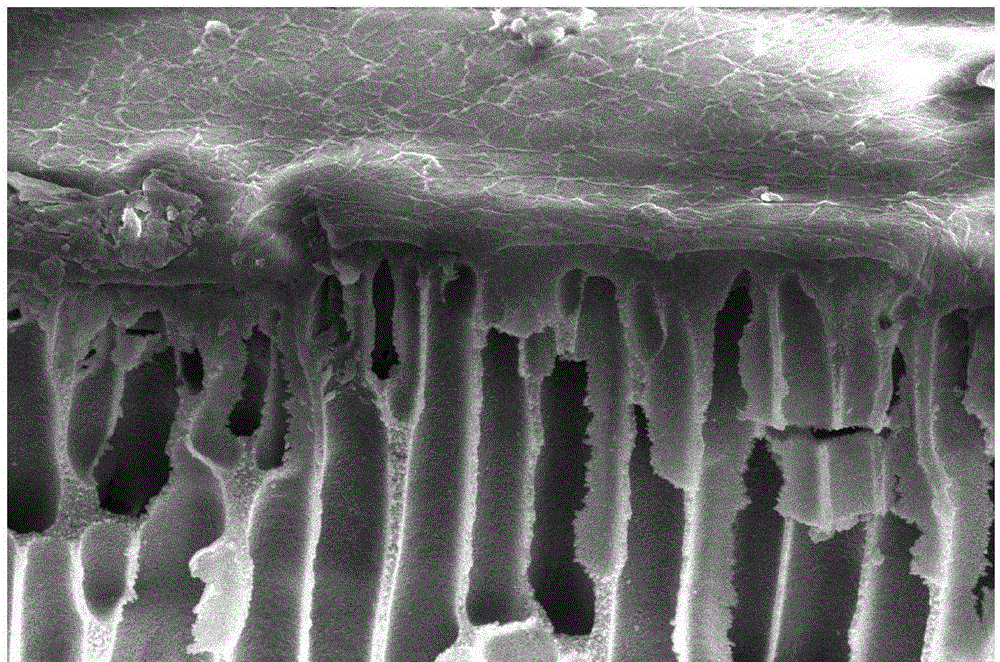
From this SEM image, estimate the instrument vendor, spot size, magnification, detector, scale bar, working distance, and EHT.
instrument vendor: FEI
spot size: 3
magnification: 4 K X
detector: ETD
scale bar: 10000 nm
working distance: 4.4 mm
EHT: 10 kV